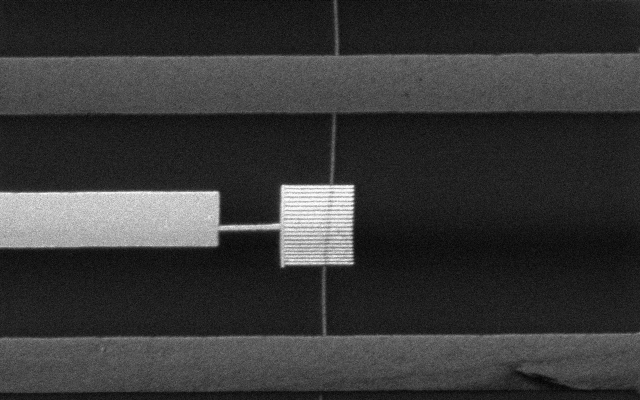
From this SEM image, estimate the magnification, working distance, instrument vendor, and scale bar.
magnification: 9 K X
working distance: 12.2 mm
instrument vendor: Hitachi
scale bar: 5000 nm